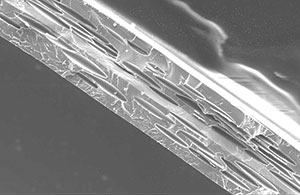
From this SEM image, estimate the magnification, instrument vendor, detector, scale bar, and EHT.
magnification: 0.05 K X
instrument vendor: JEOL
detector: SEI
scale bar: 500000 nm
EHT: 20 kV